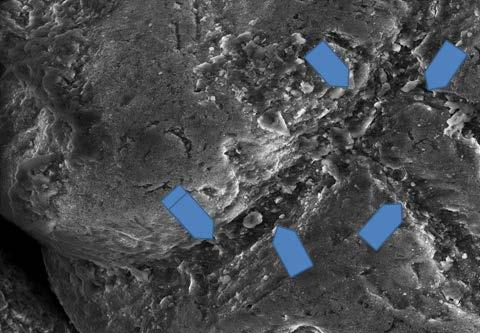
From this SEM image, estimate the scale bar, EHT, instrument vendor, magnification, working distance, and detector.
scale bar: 100000 nm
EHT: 20 kV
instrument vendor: FEI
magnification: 0.75 K X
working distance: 10 mm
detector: LFD